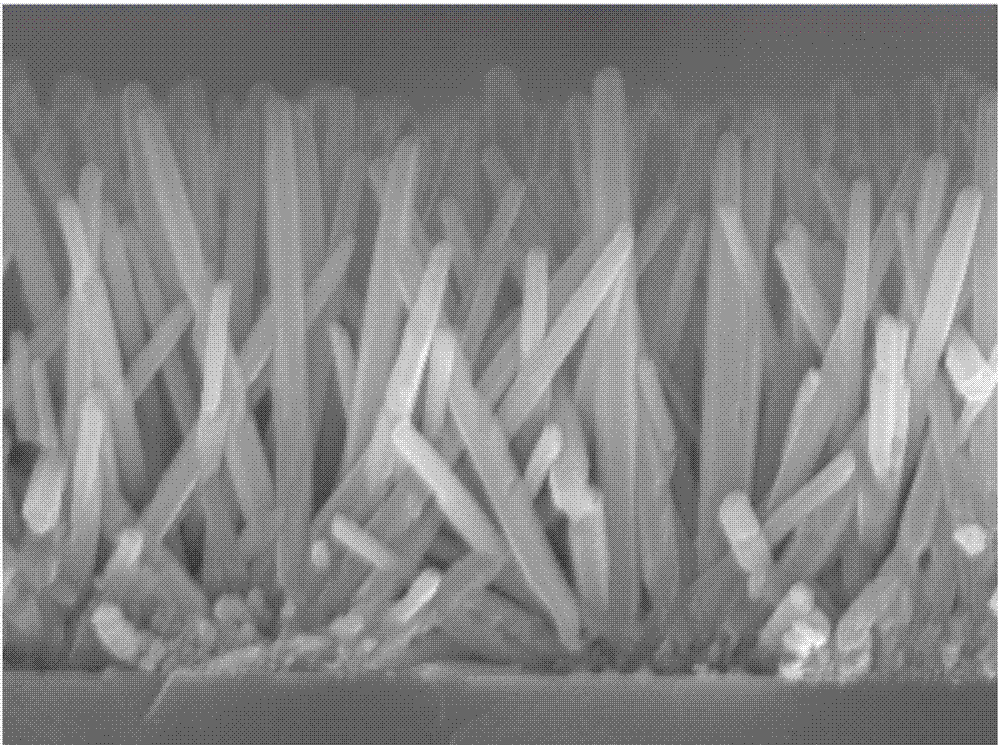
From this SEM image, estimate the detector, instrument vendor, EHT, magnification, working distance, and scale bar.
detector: SEI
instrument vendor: JEOL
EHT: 10 kV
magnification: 30 K X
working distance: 9.8 mm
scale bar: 100 nm